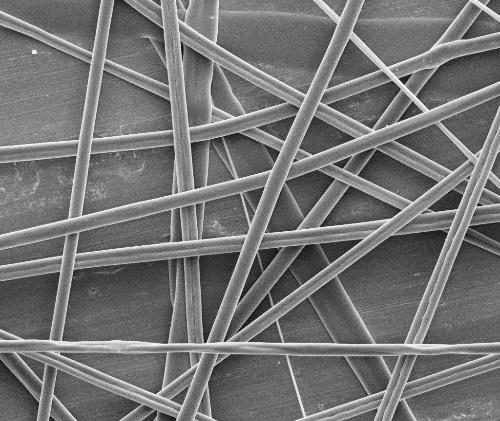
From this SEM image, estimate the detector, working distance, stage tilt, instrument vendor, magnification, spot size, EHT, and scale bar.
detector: ETD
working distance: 11.9 mm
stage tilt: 0°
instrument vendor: FEI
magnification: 5 K X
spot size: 3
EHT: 5 kV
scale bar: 10000 nm